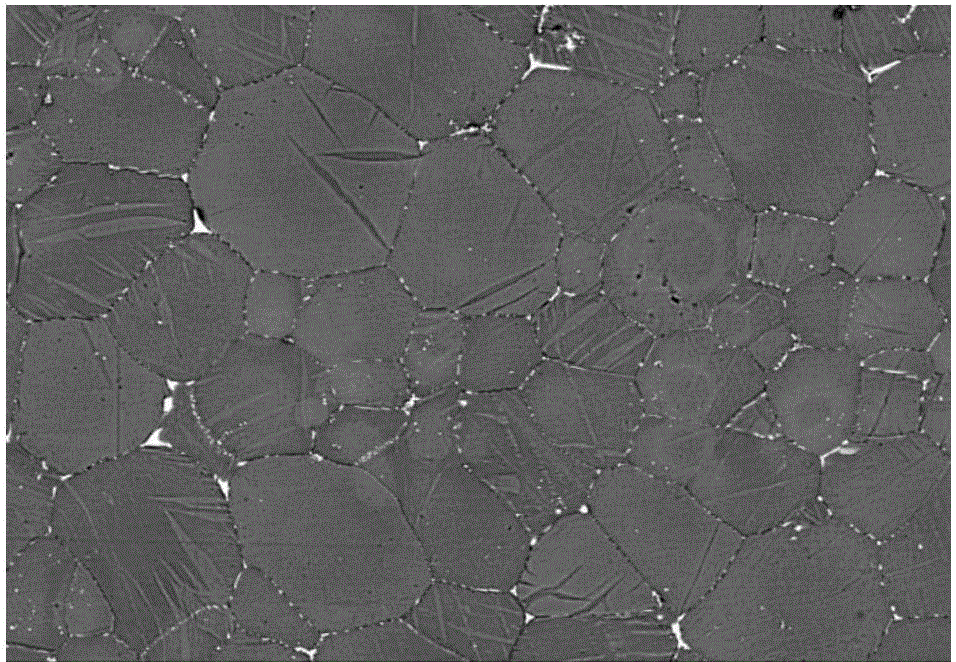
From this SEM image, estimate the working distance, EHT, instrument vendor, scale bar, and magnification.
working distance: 9.6 mm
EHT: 10 kV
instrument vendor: Hitachi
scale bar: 100000 nm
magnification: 0.5 K X